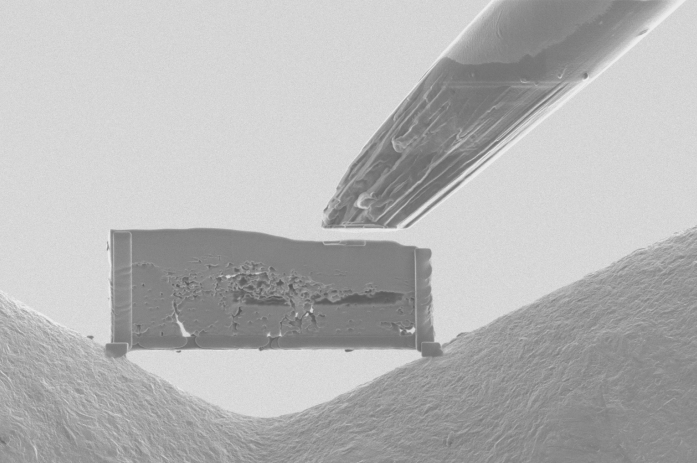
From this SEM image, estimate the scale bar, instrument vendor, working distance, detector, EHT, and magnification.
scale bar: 10000 nm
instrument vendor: FEI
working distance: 12.9 mm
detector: ICE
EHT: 30 kV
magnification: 6.49 K X